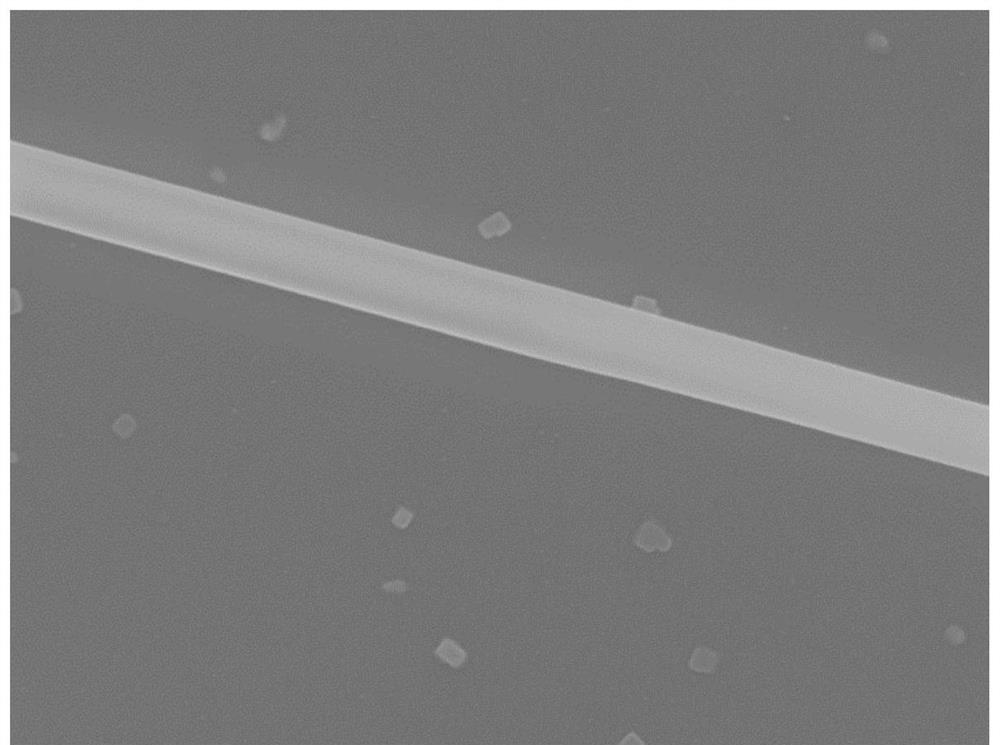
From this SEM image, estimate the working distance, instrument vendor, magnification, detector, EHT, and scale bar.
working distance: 6 mm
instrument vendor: JEOL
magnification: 13 K X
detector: SEI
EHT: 6 kV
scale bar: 1000 nm